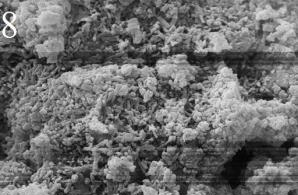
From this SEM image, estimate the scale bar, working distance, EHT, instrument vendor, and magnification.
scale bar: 1000 nm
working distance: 6.5 mm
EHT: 15 kV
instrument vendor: Zeiss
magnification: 30 K X